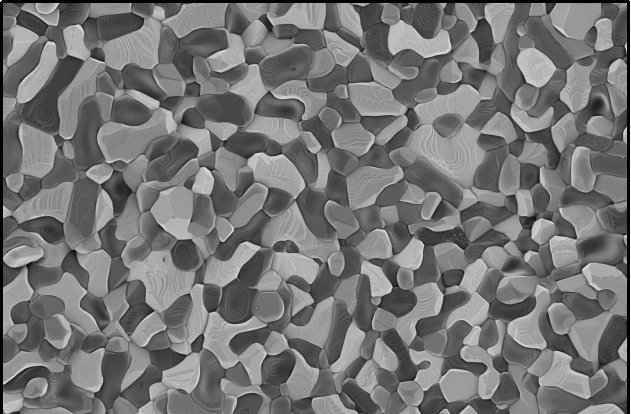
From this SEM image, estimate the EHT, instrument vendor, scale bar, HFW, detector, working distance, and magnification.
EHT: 1 kV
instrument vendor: Thermo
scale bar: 10000 nm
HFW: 25.4 µm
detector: T1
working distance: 3.6 mm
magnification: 5 K X